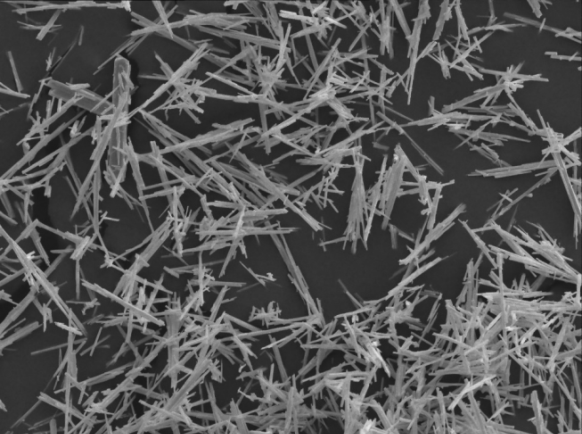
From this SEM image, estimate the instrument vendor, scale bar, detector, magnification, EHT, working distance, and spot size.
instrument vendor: other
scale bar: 6000 nm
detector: SEI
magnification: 2 K X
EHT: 20 kV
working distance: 6.07 mm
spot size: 2